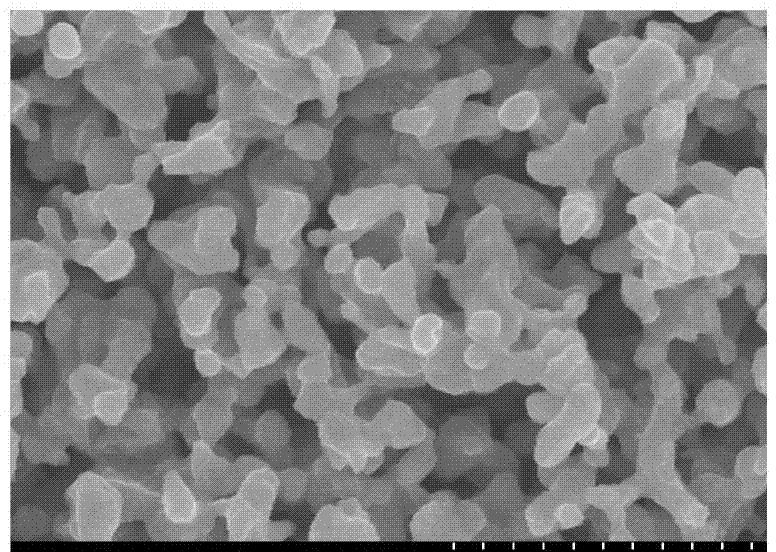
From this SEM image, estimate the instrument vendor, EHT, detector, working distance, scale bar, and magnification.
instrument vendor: Hitachi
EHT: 5 kV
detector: SE(M)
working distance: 8.4 mm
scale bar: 1000 nm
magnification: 50 K X